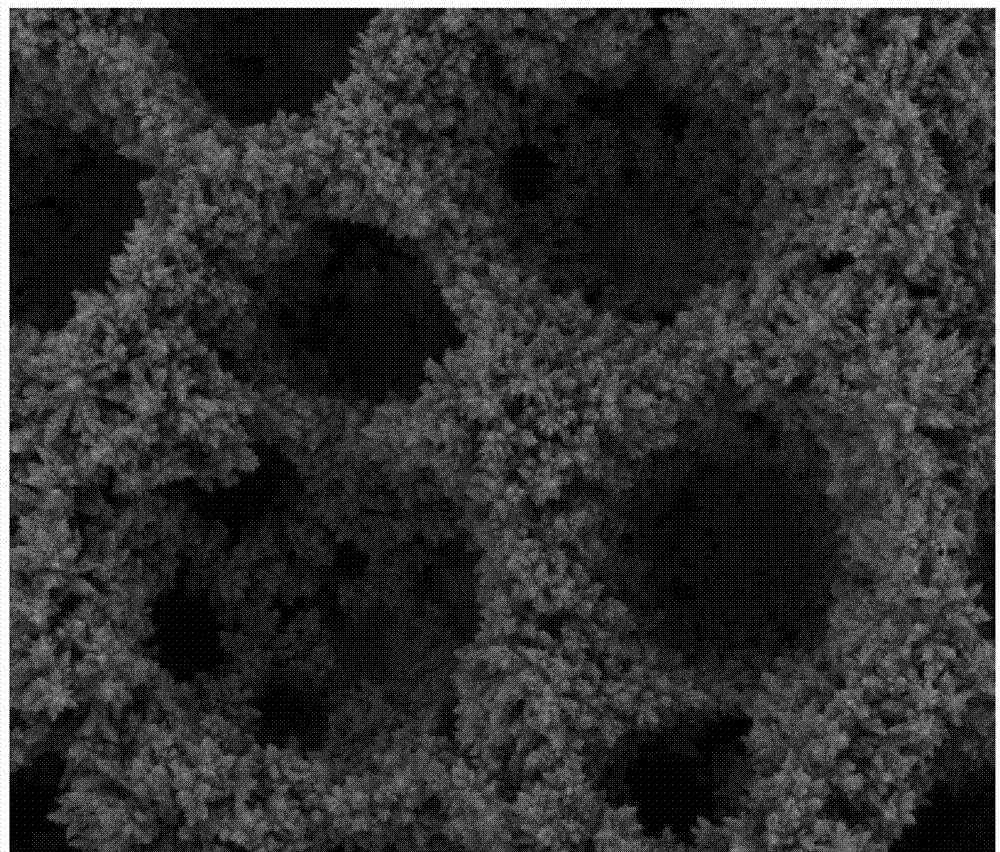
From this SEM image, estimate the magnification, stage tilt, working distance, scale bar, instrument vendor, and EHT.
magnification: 1.2 K X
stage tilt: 0°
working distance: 5.8 mm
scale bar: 50000 nm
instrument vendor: FEI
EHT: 5 kV